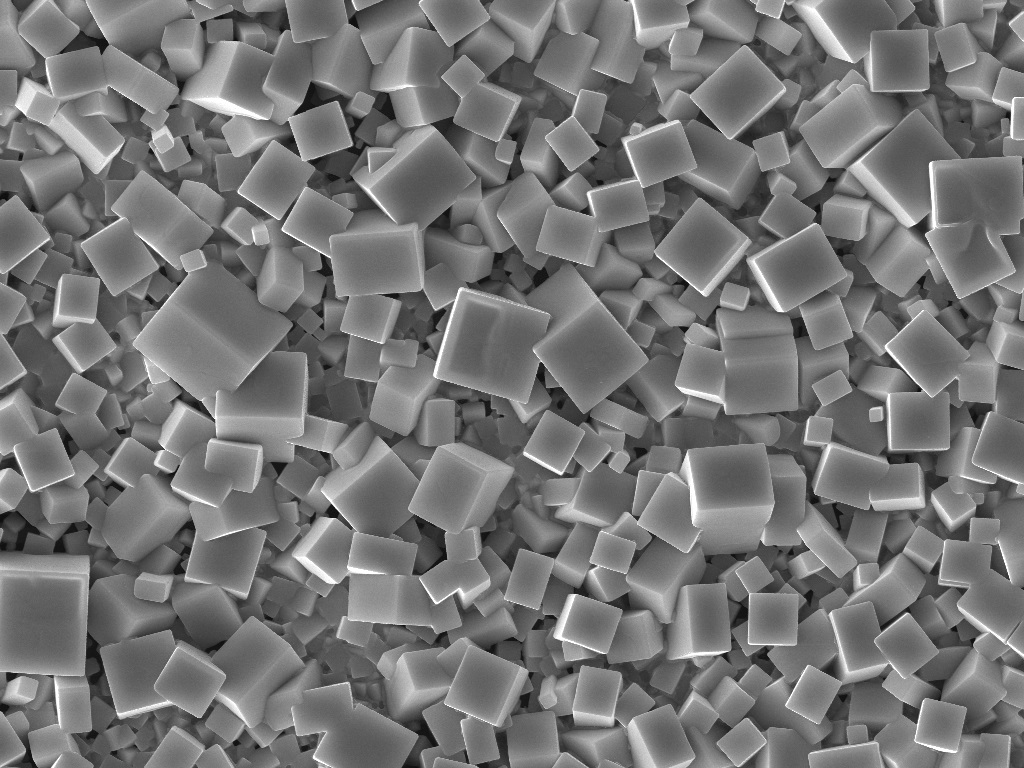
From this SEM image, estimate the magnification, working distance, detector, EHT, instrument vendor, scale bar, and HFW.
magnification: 4.5 K X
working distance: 15.05 mm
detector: SE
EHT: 20 kV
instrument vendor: Tescan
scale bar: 10000 nm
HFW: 61.6 µm